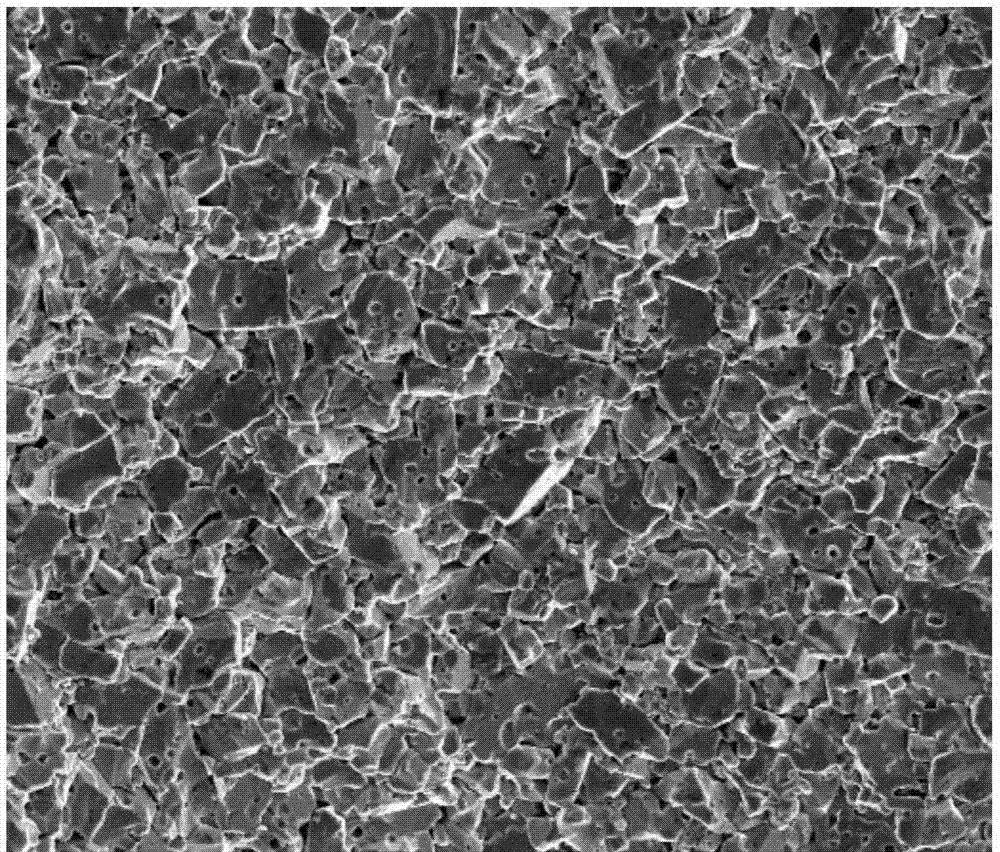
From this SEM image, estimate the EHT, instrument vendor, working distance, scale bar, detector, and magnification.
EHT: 20 kV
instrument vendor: FEI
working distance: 6.9 mm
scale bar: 50000 nm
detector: SE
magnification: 1 K X